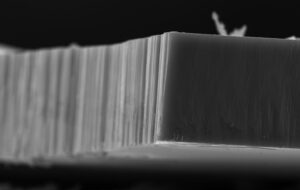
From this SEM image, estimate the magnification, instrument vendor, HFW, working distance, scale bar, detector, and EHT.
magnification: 5 K X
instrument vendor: FEI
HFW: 82.9 µm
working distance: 4.9 mm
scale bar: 20000 nm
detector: ETD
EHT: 20 kV